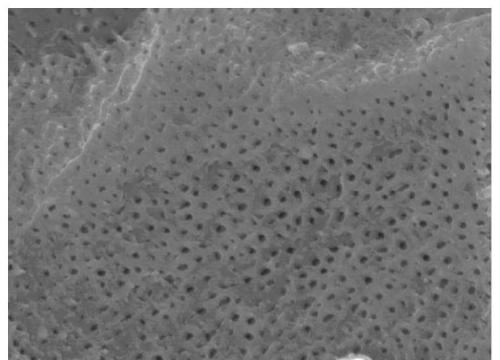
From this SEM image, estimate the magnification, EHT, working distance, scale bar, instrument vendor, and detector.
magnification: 20 K X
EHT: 10 kV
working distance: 9.7 mm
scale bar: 1000 nm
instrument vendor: JEOL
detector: SEI